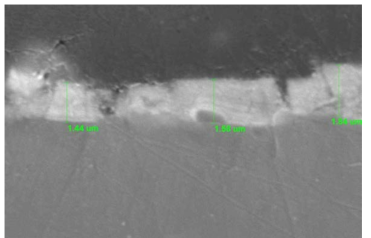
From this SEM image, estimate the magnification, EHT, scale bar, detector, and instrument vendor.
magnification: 10 K X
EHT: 20 kV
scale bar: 1000 nm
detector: SEI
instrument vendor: JEOL